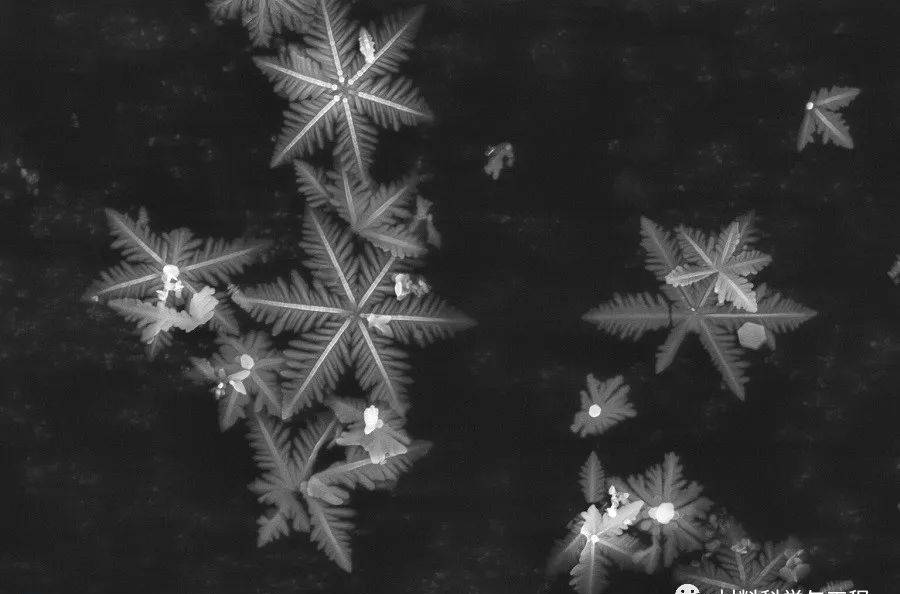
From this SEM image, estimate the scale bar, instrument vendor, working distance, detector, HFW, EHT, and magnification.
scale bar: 10000 nm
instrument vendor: Thermo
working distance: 10.4 mm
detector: ETD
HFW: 27.6 µm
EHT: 15 kV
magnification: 15 K X